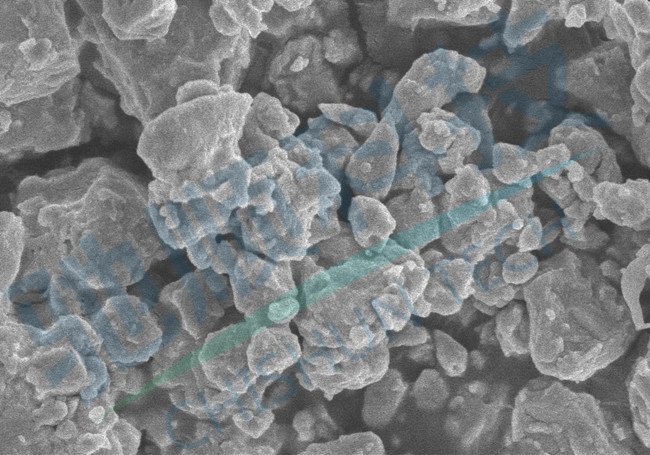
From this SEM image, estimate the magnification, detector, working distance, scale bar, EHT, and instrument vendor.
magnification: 30 K X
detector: SE(UL)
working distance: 8.8 mm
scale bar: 1000 nm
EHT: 3 kV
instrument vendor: Hitachi